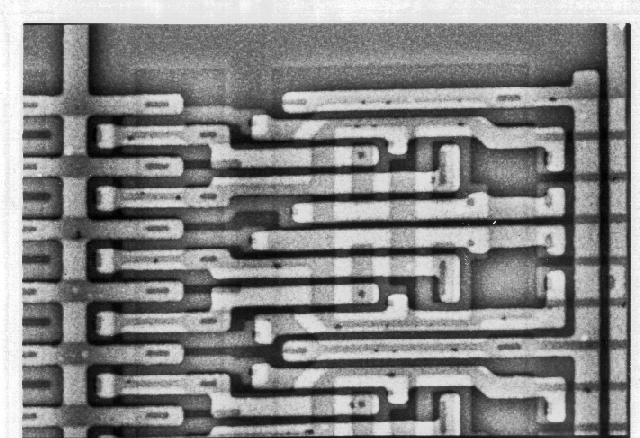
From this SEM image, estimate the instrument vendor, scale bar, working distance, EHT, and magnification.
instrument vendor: JEOL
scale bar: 10000 nm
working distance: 25 mm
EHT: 40 kV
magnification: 0.65 K X